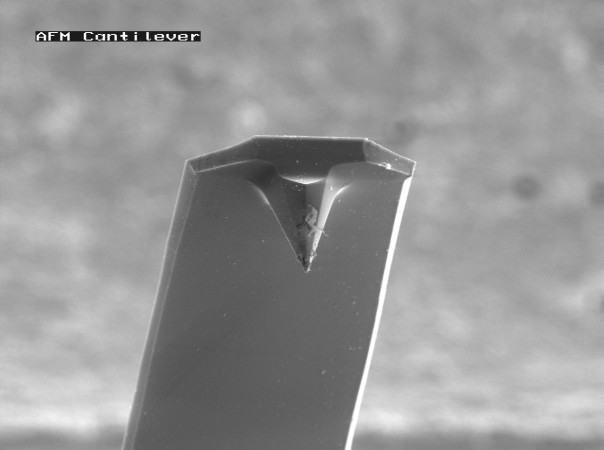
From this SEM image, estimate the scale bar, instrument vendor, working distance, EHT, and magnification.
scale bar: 20000 nm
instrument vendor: JEOL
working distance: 22 mm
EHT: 8 kV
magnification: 1 K X